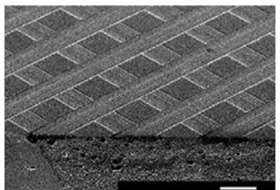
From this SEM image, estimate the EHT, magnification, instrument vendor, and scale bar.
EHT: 25 kV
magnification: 0.1 K X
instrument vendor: JEOL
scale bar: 200000 nm